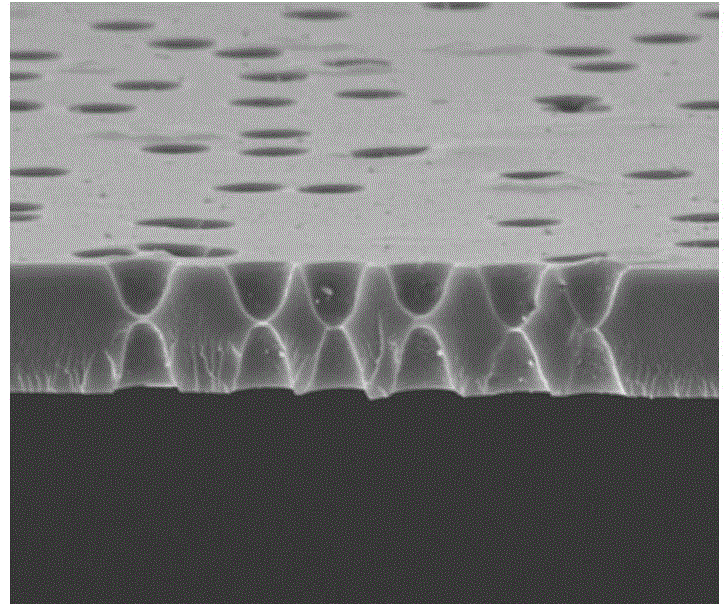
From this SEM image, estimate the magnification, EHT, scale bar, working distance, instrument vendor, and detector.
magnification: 8 K X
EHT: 10 kV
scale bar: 10000 nm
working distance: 6.3 mm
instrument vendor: FEI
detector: ETD